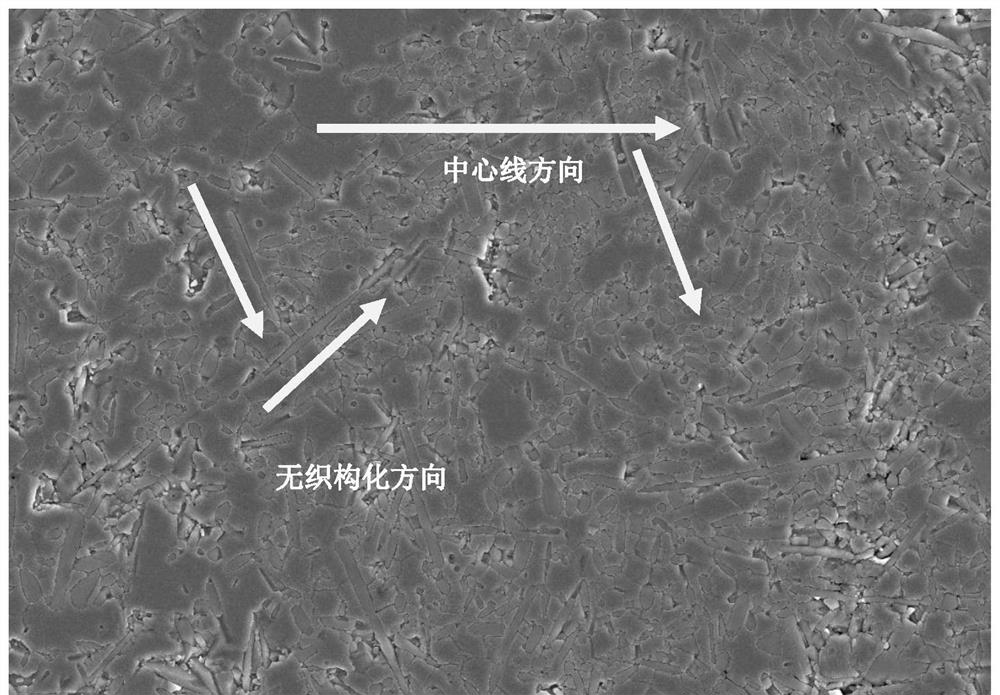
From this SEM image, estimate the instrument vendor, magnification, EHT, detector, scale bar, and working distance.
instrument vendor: Hitachi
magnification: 5 K X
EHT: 10 kV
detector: SE(UL)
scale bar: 20000 nm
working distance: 12.7 mm